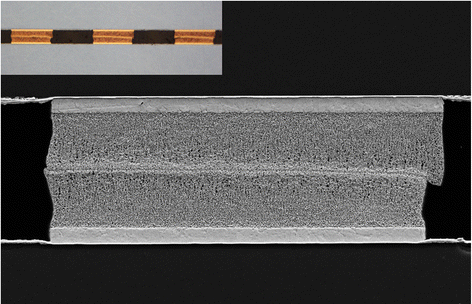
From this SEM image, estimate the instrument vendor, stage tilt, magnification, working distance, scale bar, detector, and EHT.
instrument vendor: Zeiss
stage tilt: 0°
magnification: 1.5 K X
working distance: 8 mm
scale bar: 10000 nm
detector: SE2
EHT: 10 kV